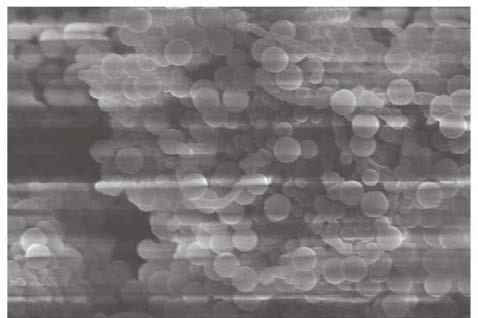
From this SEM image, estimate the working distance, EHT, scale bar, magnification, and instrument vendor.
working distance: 12.4 mm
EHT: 10 kV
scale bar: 5000 nm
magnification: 10 K X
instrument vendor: Hitachi